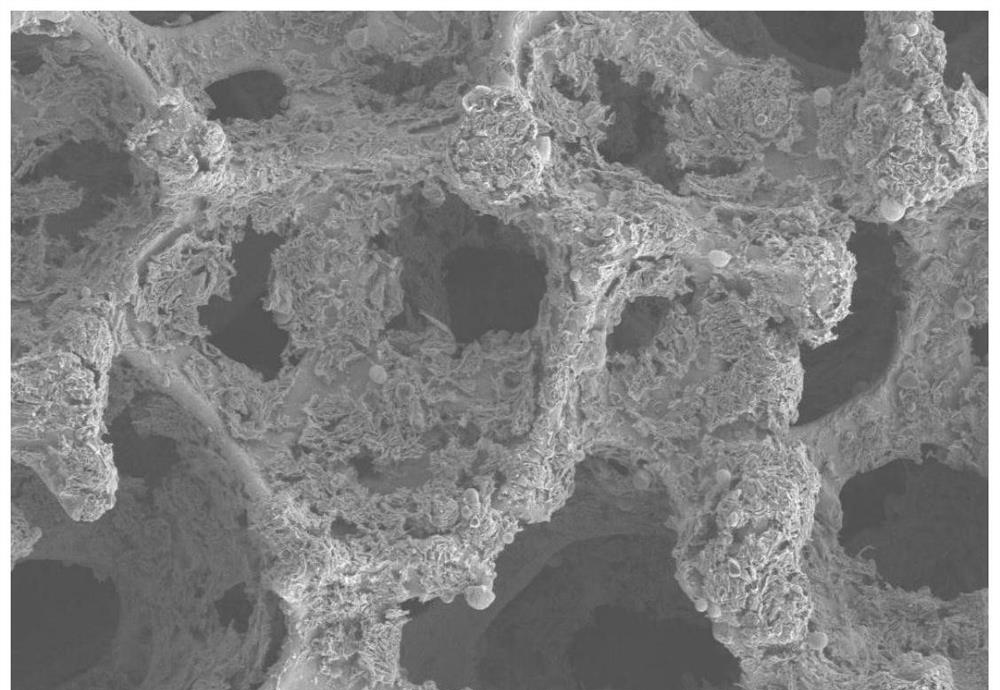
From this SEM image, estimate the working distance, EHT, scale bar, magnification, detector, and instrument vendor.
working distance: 8.6 mm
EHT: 10 kV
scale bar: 100000 nm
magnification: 0.1 K X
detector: SE2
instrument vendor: Zeiss